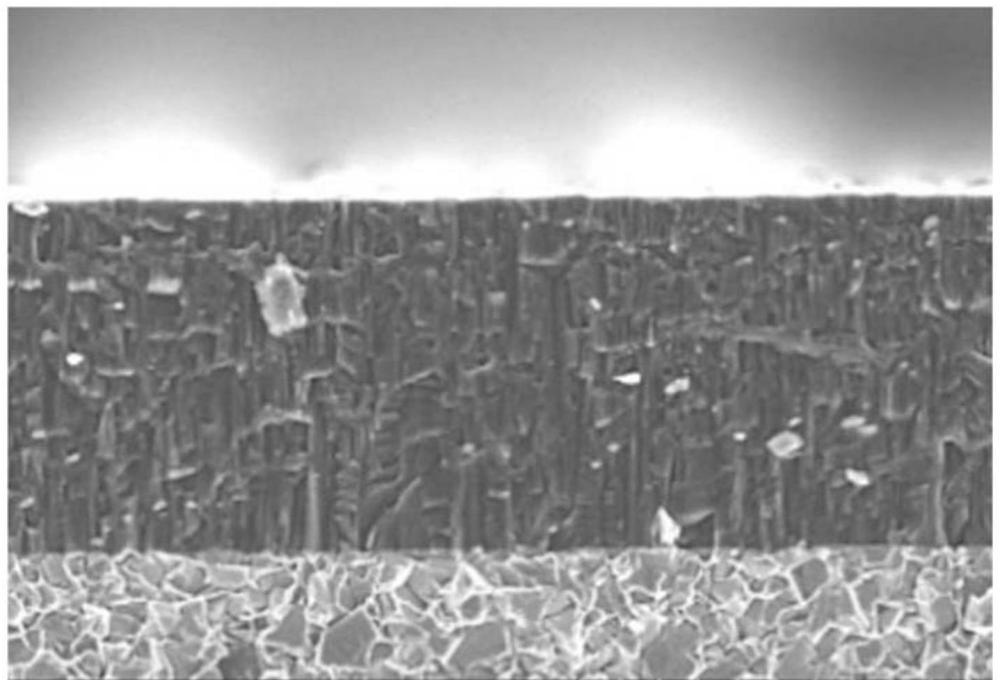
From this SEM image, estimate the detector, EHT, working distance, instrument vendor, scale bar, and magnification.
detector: SE(L)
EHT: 5 kV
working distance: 13 mm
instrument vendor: Hitachi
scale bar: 5000 nm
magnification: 10 K X